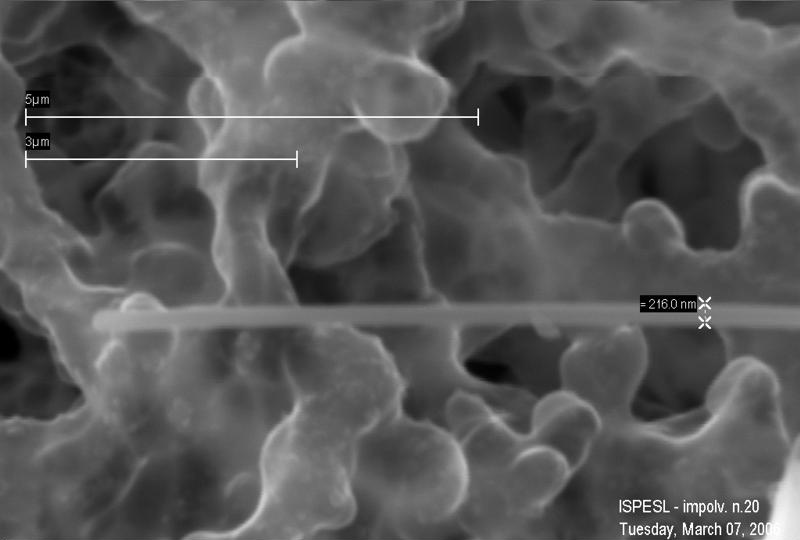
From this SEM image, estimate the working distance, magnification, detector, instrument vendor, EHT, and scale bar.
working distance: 21 mm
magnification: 43.86 K X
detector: SE1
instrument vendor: Zeiss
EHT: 25 kV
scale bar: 1000 nm